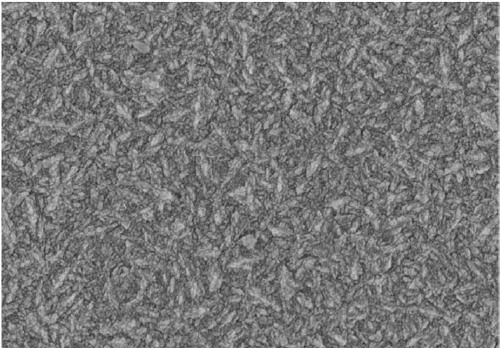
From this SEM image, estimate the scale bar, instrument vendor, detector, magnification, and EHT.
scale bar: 2000 nm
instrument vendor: Hitachi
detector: SE(U)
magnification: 25 K X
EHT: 4 kV